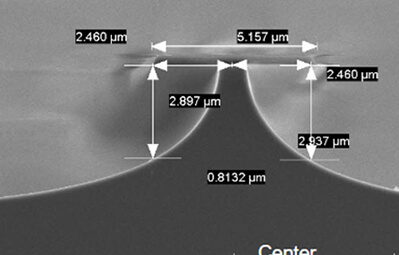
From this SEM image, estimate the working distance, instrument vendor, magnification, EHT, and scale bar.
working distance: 10.5 mm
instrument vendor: Hitachi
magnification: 10 K X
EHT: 5 kV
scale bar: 5000 nm